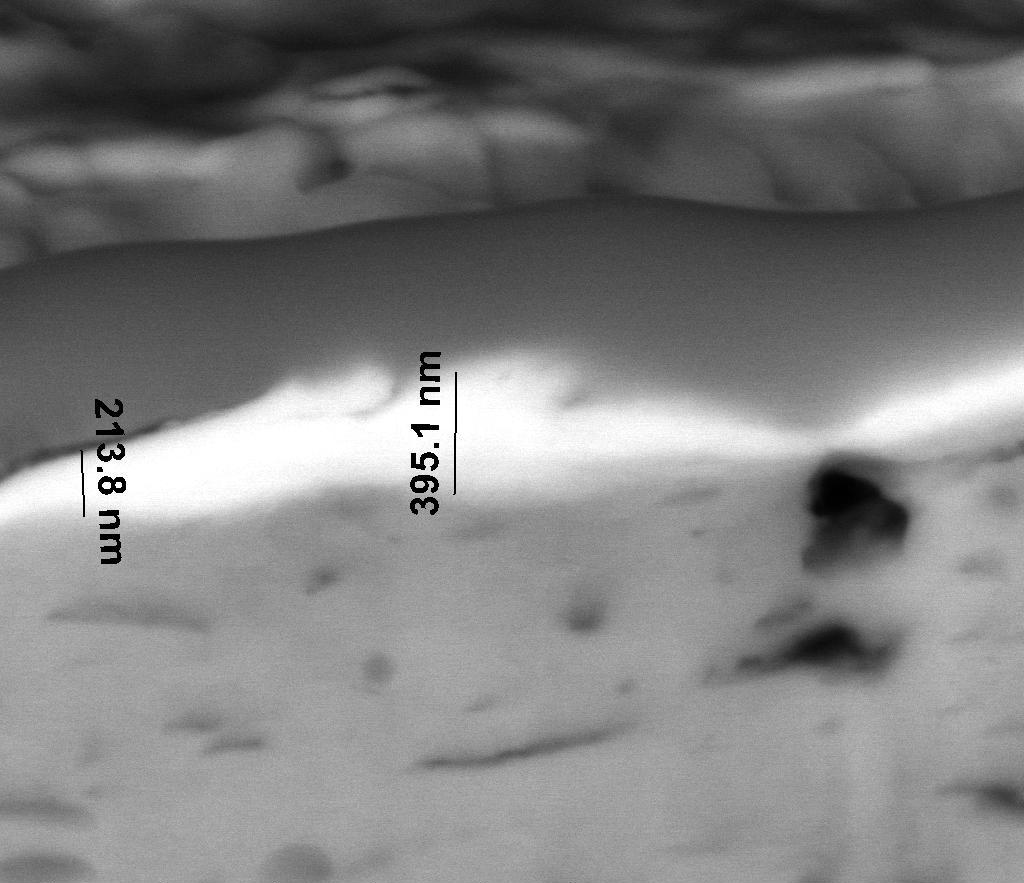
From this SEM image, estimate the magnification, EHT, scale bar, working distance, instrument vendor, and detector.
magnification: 45 K X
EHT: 20 kV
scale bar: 1000 nm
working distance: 10 mm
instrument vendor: FEI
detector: BSED